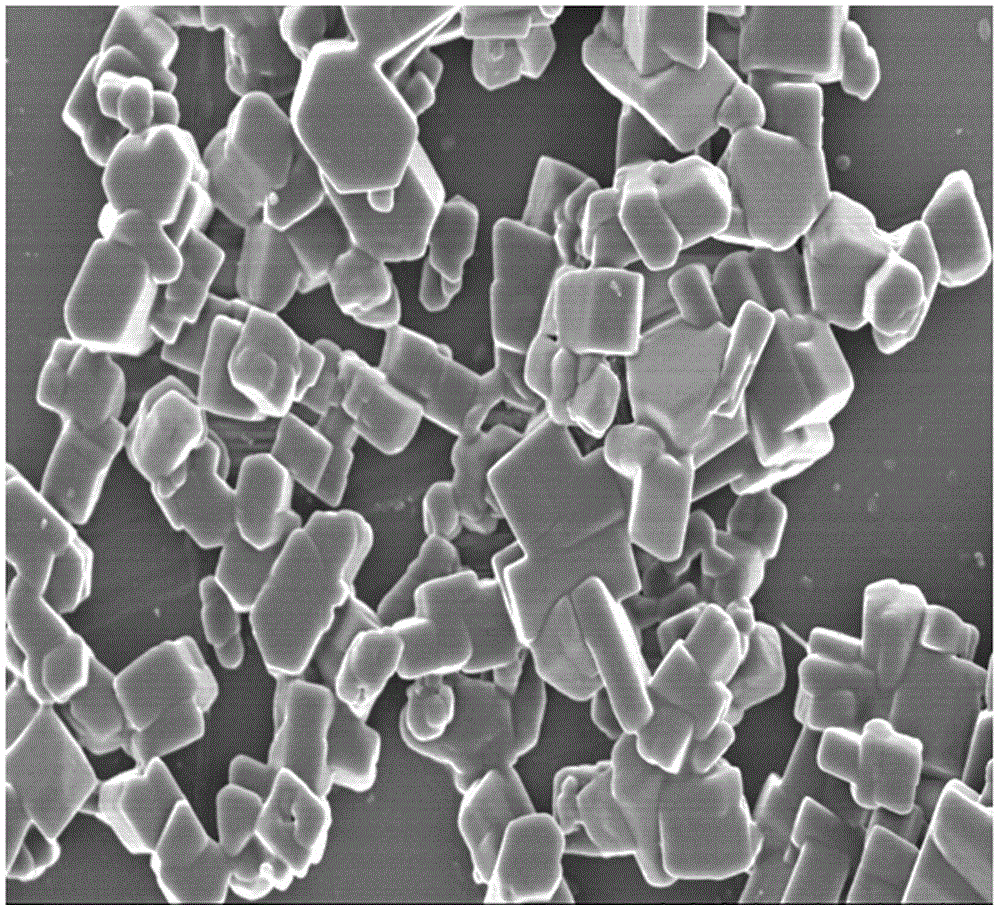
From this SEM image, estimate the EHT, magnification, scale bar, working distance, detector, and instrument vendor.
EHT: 3 kV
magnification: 8 K X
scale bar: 5000 nm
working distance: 9.5 mm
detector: SE(M)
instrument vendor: Hitachi